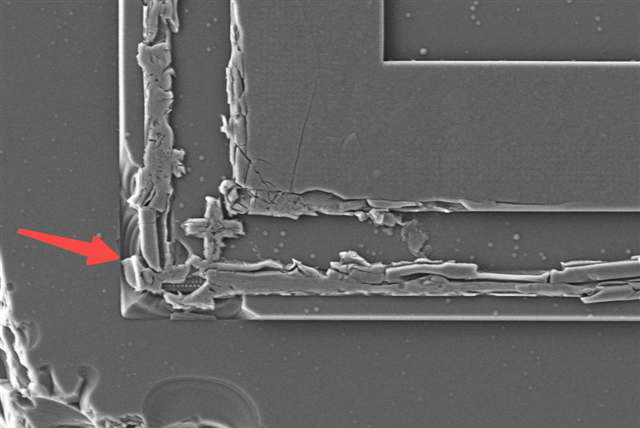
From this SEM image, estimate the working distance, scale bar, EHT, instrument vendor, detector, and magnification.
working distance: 6.6 mm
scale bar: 10000 nm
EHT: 10 kV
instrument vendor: Zeiss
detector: SE2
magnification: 1 K X